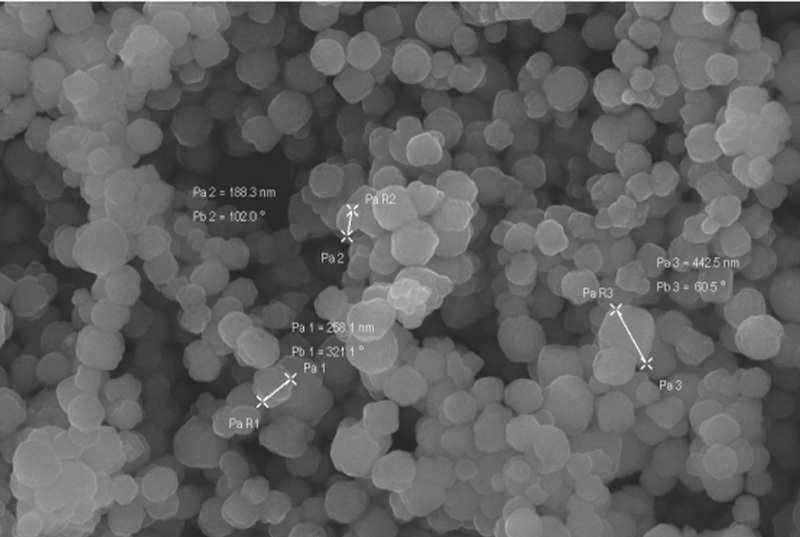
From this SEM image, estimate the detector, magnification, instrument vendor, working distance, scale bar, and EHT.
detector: InLens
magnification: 20 K X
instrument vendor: Zeiss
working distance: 6.8 mm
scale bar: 200 nm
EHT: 15 kV